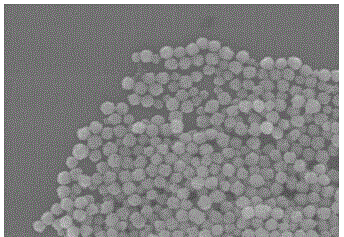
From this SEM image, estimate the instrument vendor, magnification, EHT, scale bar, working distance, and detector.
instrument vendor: Hitachi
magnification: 45 K X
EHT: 10 kV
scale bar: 1000 nm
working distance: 11.3 mm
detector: SE(M,LA0)